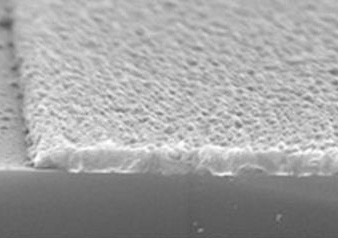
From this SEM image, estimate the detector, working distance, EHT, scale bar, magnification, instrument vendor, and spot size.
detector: SE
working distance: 9.6 mm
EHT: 15 kV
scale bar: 1000 nm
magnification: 20 K X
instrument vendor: FEI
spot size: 3